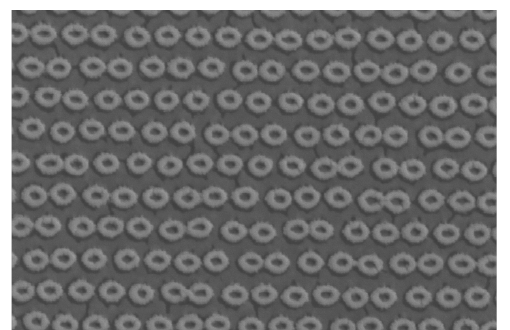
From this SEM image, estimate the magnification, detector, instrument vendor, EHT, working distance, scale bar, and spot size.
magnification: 90.296 K X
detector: TLD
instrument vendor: FEI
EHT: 10 kV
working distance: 5 mm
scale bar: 1000 nm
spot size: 3